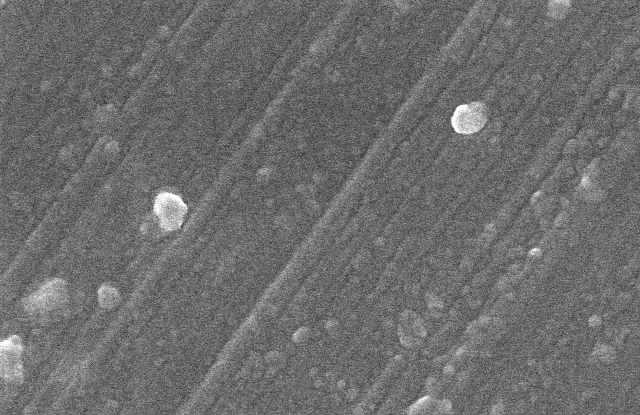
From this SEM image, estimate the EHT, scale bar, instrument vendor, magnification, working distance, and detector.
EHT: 10 kV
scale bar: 1000 nm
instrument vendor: Hitachi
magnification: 50 K X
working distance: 8.2 mm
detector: SE(U)